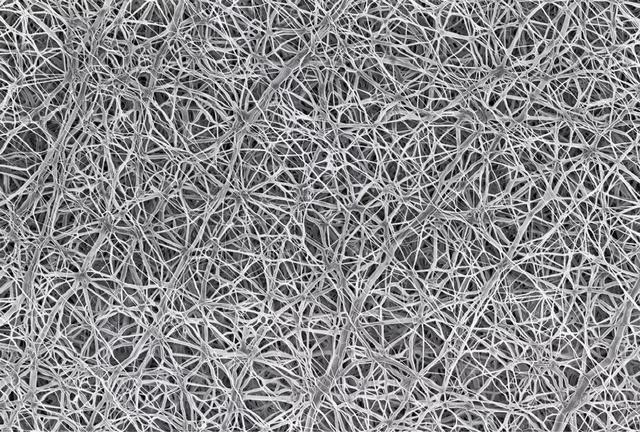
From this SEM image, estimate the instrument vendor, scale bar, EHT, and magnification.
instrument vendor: Zeiss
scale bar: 1000 nm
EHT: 2 kV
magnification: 5 K X